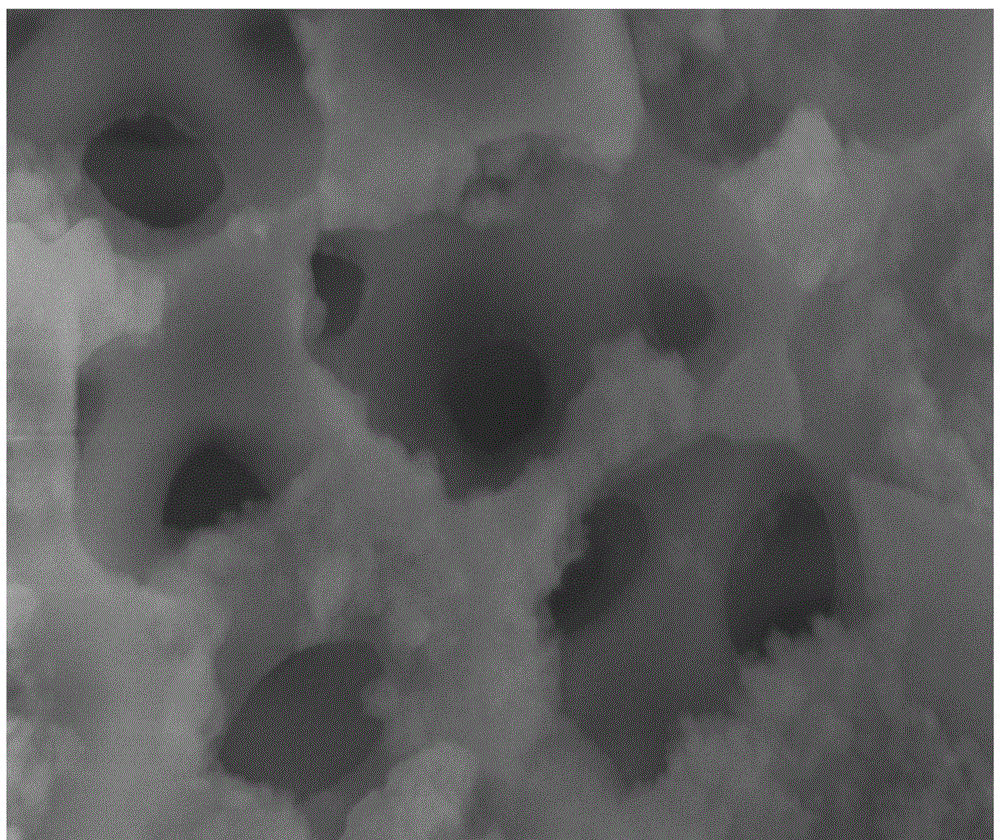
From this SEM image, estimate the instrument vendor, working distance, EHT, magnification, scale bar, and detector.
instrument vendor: Hitachi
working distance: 7.9 mm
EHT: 15 kV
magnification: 30 K X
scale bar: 1000 nm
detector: SE(M)